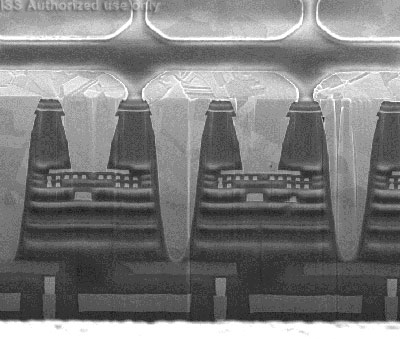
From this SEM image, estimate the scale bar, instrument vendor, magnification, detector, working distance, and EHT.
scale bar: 5000 nm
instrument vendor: FEI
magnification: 12 K X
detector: TLD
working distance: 4.1 mm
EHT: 5 kV